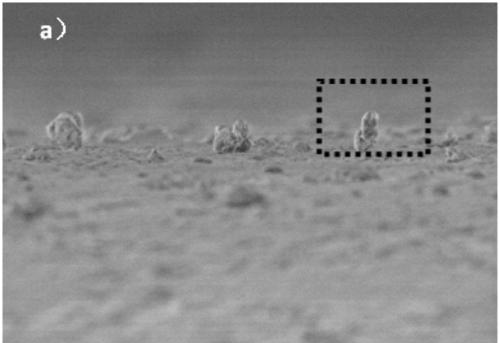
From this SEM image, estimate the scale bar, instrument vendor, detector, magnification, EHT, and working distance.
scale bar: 10000 nm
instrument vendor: Hitachi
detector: SE(UL)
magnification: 4.5 K X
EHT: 3 kV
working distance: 9.1 mm